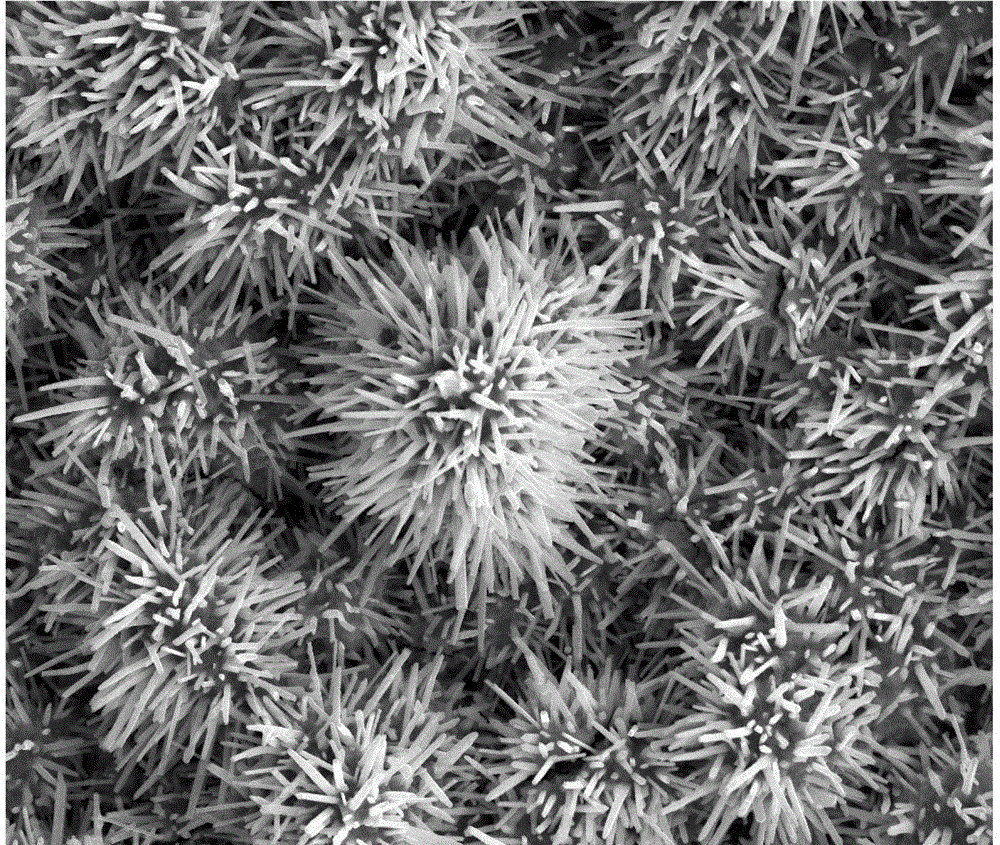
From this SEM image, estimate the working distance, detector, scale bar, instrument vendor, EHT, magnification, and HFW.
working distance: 9.3 mm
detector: ETD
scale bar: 10000 nm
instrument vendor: FEI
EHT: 20 kV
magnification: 2.5 K X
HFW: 54.08 µm